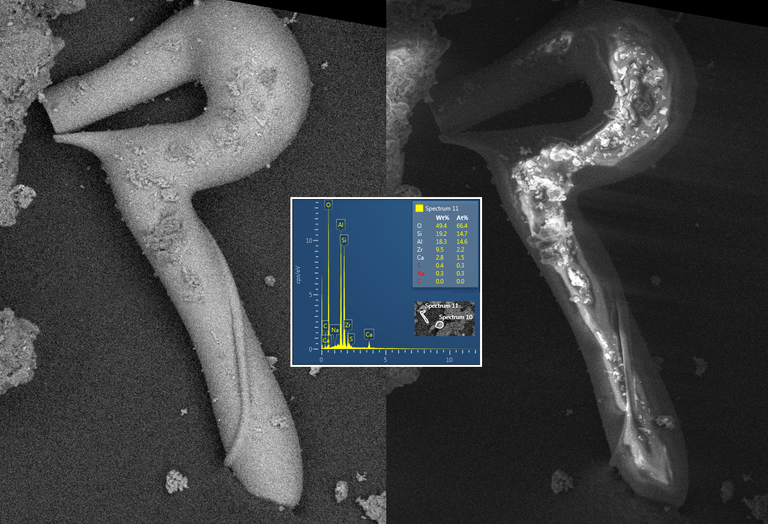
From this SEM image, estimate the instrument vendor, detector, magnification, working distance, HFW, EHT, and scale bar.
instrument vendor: Tescan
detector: BSE, SE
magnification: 1 K X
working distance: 14.97 mm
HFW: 277 µm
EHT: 15 kV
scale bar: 200000 nm